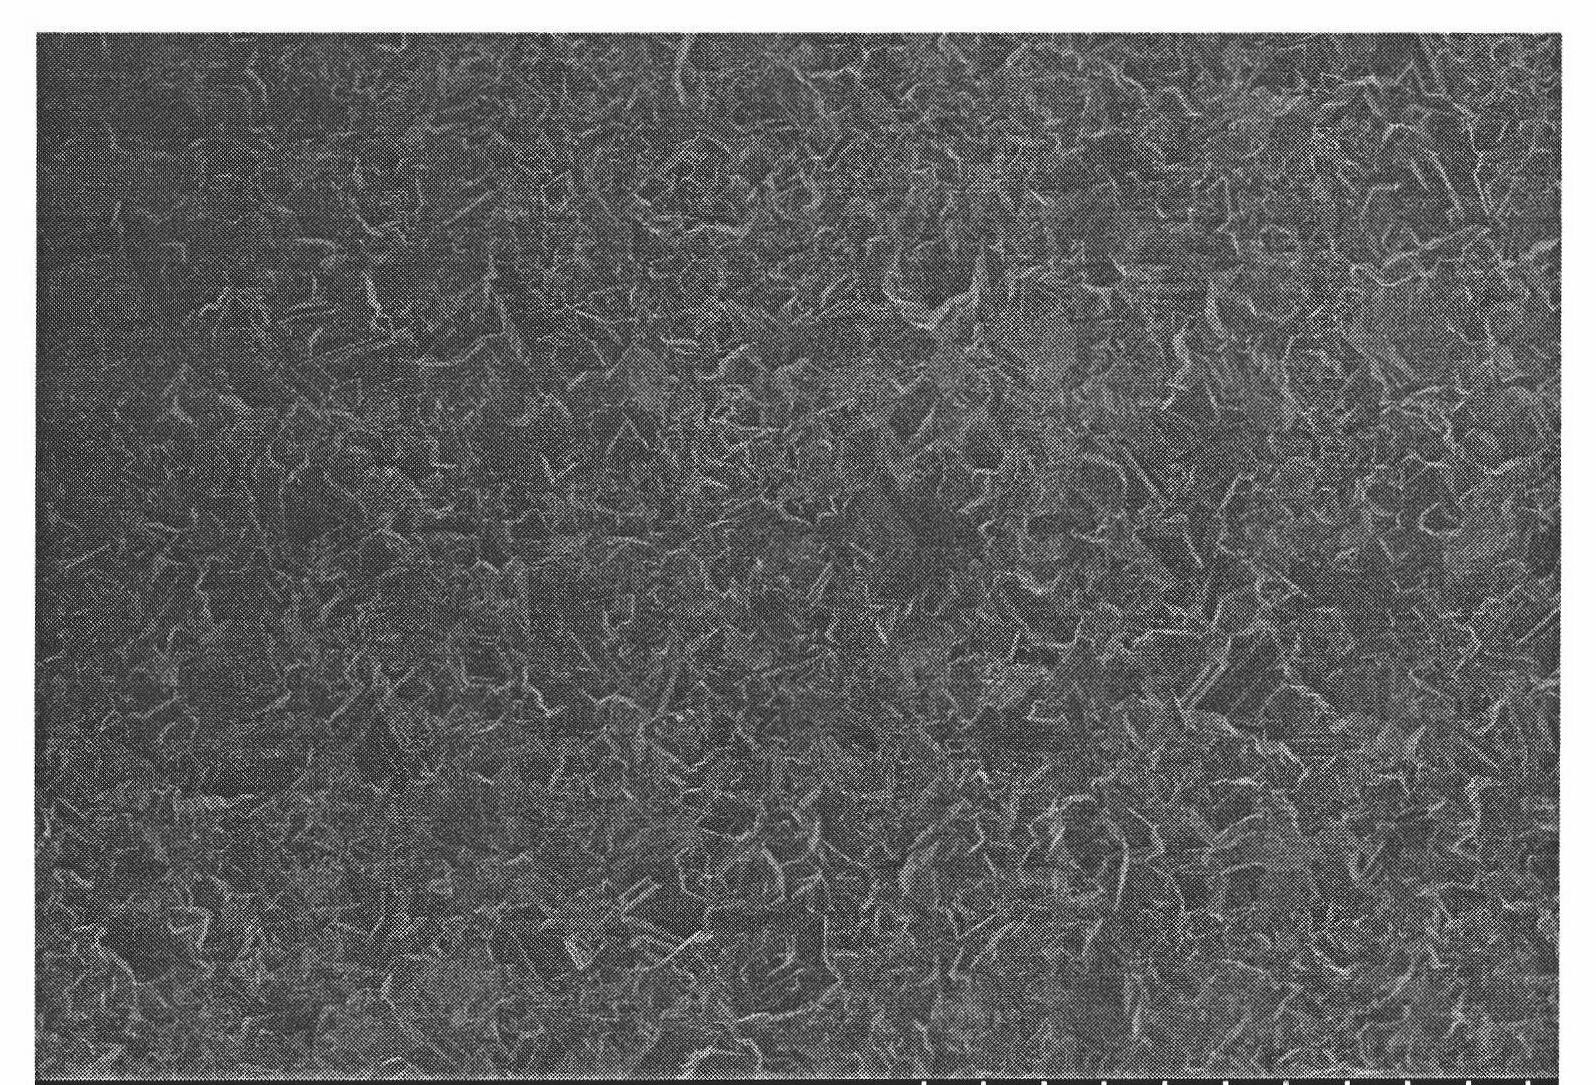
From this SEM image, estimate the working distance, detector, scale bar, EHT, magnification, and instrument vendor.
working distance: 4.2 mm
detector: SE(U)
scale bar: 50000 nm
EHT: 5 kV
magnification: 1 K X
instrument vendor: Hitachi